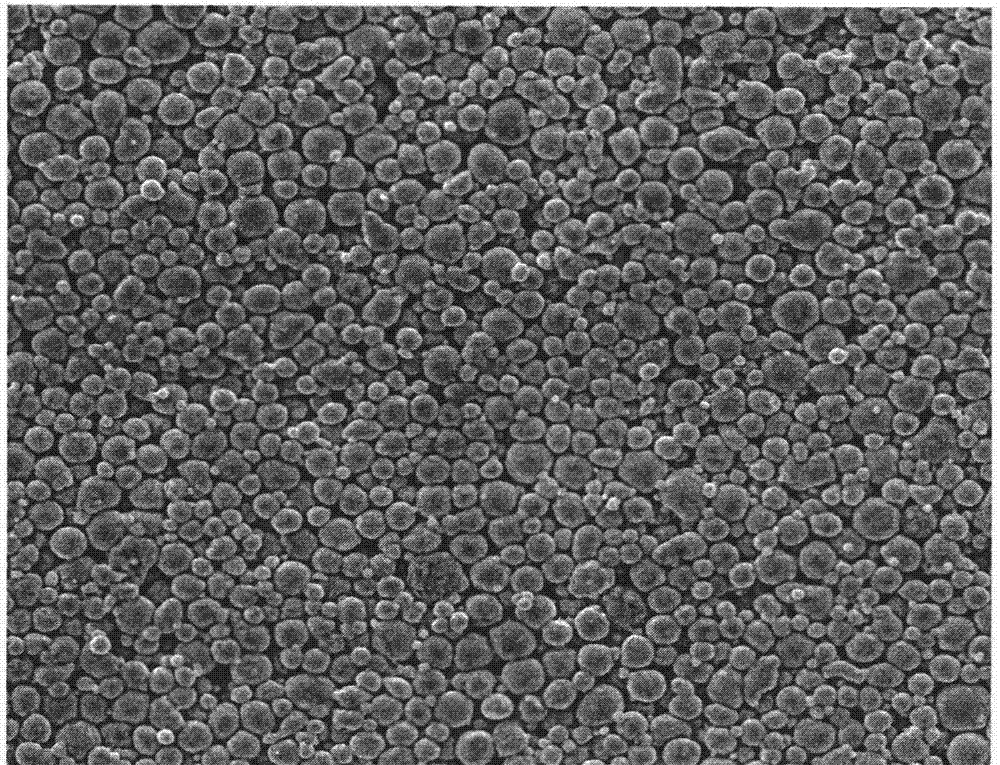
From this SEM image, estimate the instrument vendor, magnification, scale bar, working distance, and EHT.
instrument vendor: FEI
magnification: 1 K X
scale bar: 50000 nm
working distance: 10 mm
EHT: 20 kV